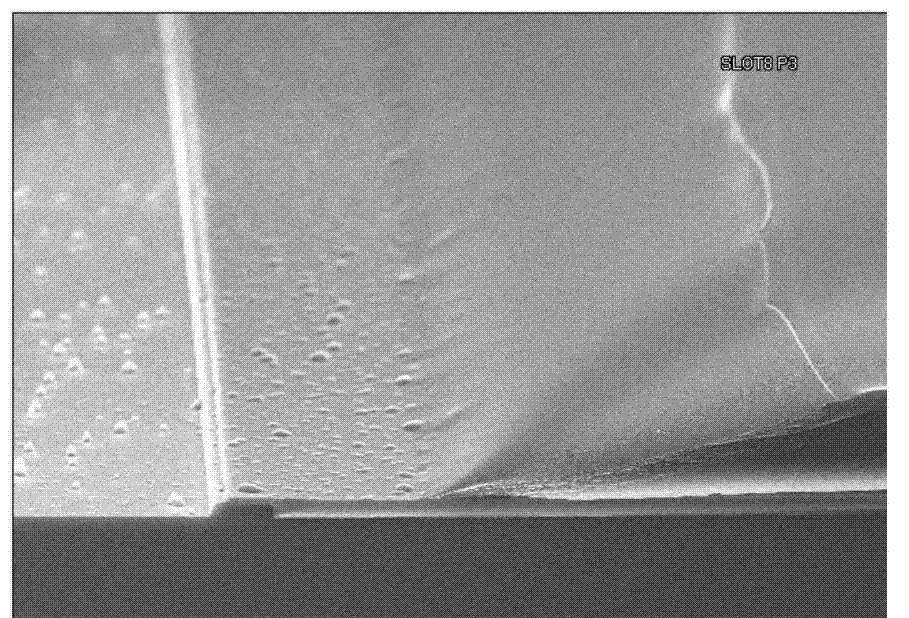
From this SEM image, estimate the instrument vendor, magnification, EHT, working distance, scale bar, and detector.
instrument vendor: Hitachi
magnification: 1.5 K X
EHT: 15 kV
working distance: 8.2 mm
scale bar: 30000 nm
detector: SE(U)